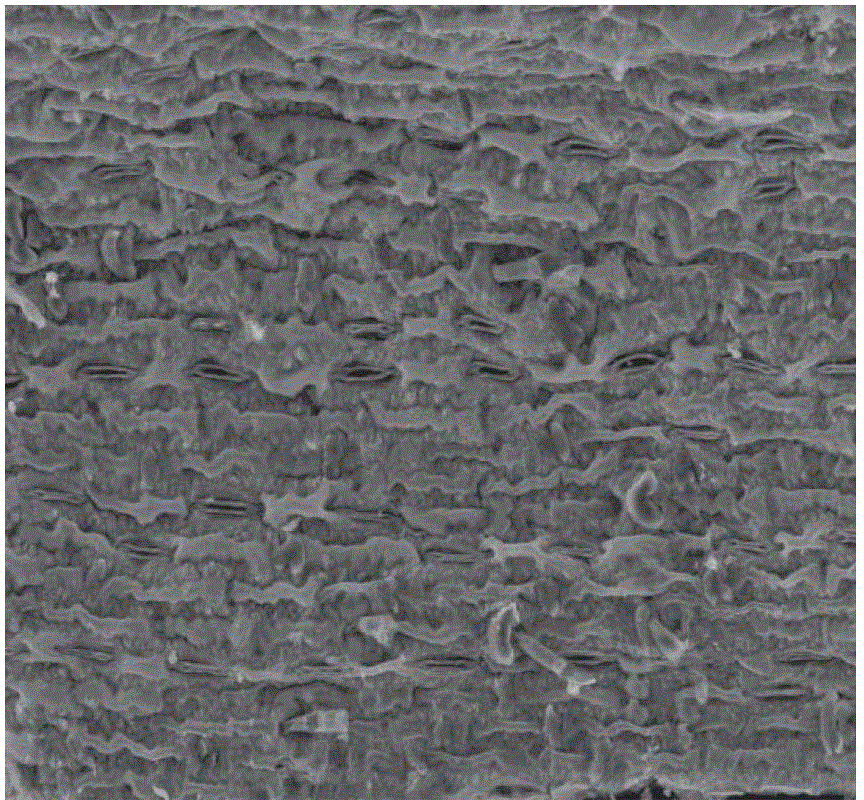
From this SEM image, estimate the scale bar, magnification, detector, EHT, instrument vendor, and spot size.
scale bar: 100000 nm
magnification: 0.8 K X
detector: ETD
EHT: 20 kV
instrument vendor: FEI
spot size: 3